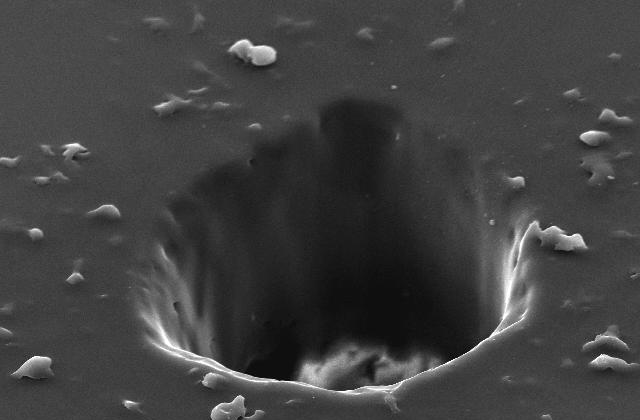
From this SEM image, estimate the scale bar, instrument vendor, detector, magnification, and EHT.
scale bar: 20000 nm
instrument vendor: JEOL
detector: SEI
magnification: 0.6 K X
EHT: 20 kV